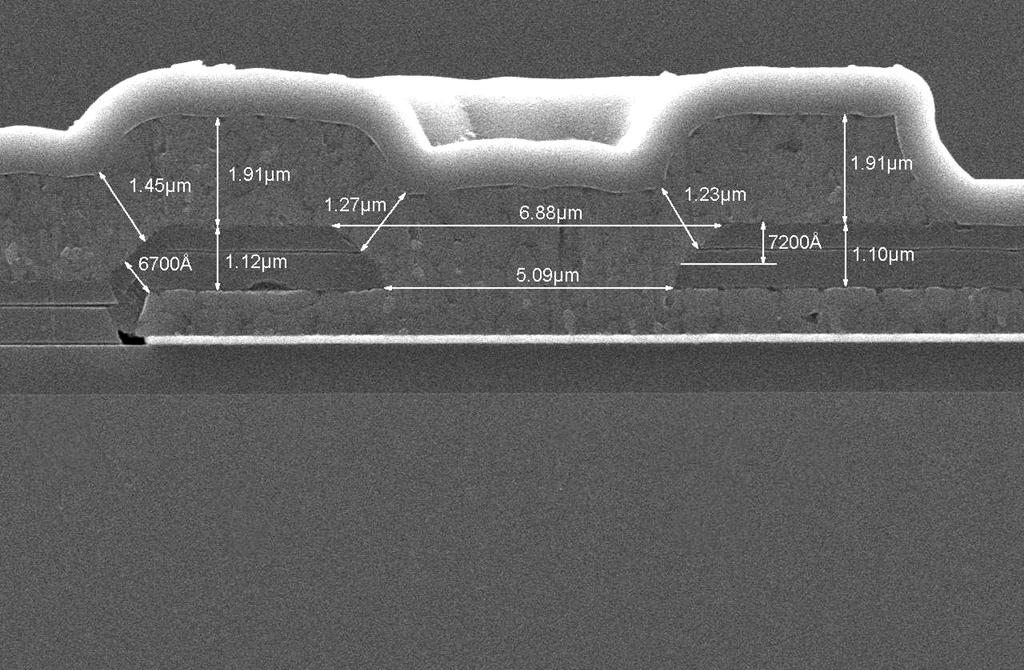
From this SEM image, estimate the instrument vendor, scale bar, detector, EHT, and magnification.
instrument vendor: JEOL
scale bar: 2000 nm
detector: SEI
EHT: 10 kV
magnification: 7 K X